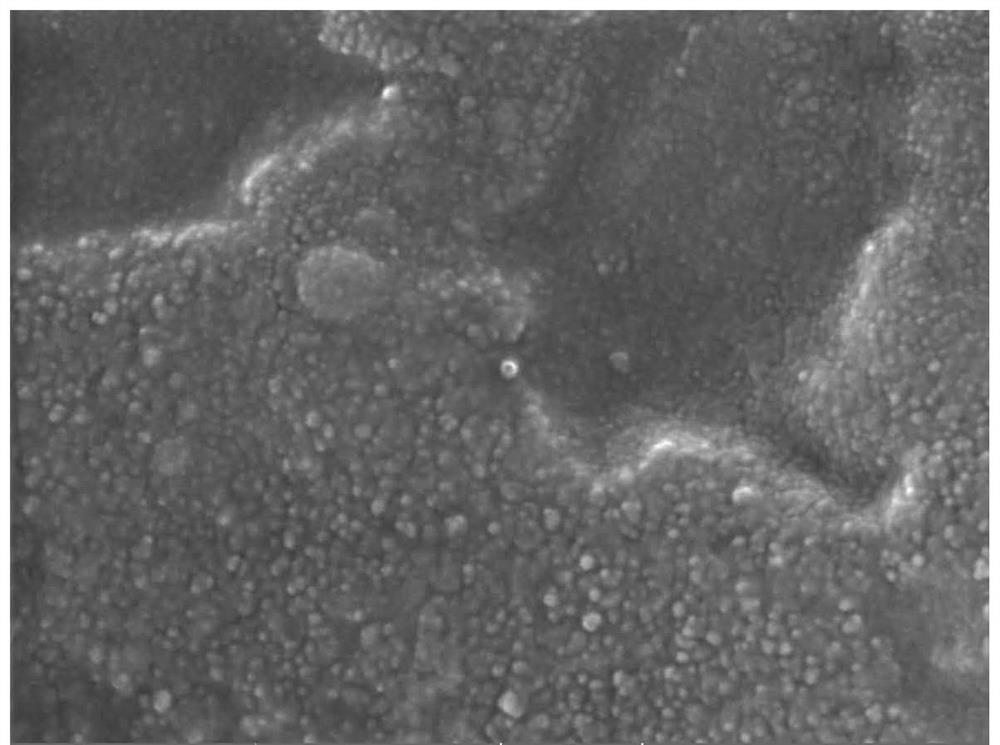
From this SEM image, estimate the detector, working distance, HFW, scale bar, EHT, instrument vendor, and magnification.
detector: In-Beam SE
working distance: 4.95 mm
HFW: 1.39 µm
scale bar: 200 nm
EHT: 20 kV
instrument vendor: Tescan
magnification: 199 K X